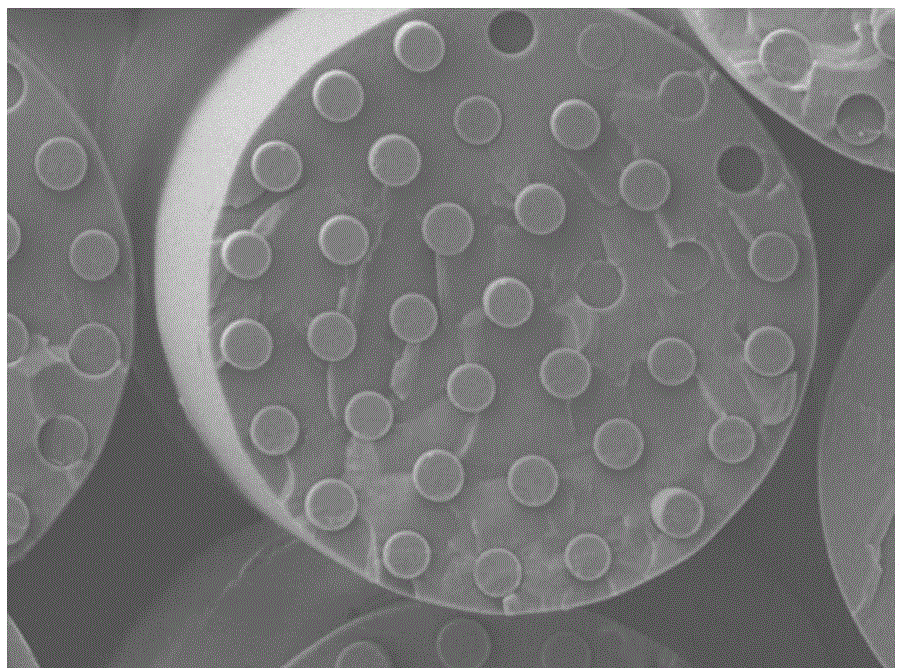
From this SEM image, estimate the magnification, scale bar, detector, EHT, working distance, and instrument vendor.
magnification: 5 K X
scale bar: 1000 nm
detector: SEI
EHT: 10 kV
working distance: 8 mm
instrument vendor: JEOL